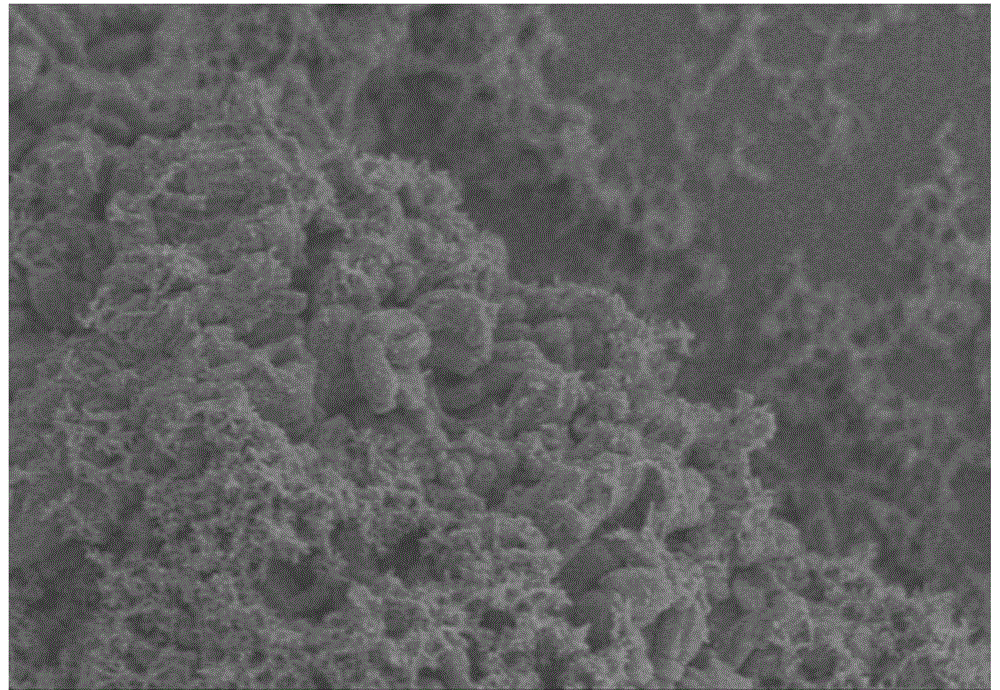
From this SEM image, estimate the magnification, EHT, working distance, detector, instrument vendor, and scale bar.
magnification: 10 K X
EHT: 2 kV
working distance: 7.4 mm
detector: SE(M)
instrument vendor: Hitachi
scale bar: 5000 nm